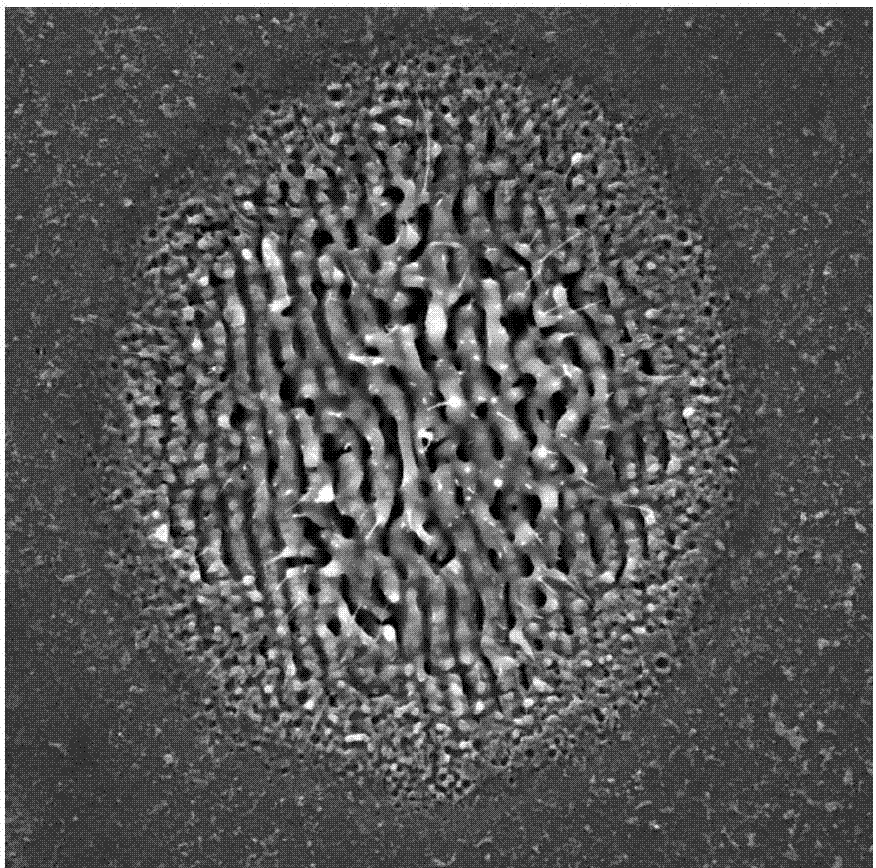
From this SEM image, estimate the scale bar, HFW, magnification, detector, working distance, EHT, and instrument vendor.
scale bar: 5000 nm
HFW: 23.1 µm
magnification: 9 K X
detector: SE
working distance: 8.73 mm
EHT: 10 kV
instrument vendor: Tescan